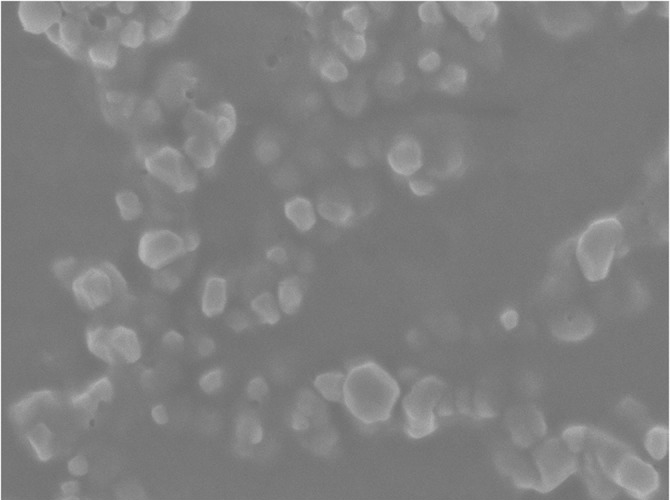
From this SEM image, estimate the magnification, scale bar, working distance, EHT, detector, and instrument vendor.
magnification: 30 K X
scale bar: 100 nm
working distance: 7 mm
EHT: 3 kV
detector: SEI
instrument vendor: JEOL